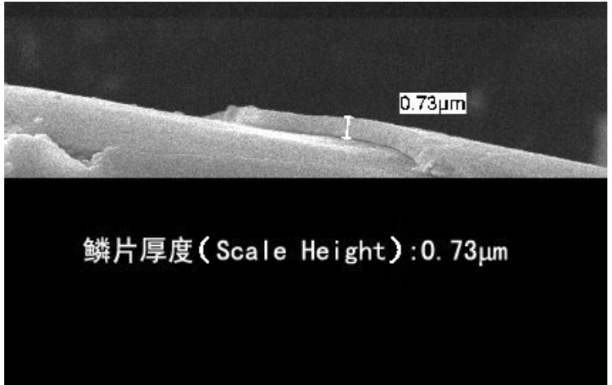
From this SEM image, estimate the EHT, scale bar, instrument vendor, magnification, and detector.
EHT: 10 kV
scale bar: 2000 nm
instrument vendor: JEOL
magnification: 6 K X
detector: SEI